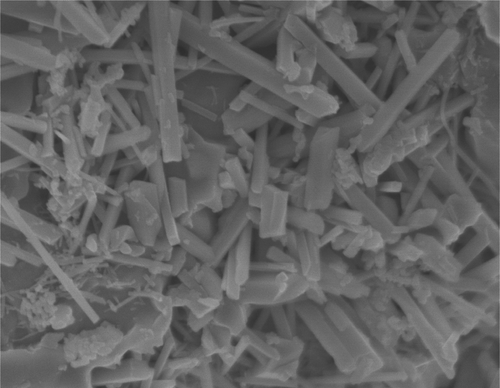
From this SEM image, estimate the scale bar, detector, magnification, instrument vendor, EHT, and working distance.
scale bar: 100 nm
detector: SEI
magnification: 45 K X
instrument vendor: JEOL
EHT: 5 kV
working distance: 4.5 mm